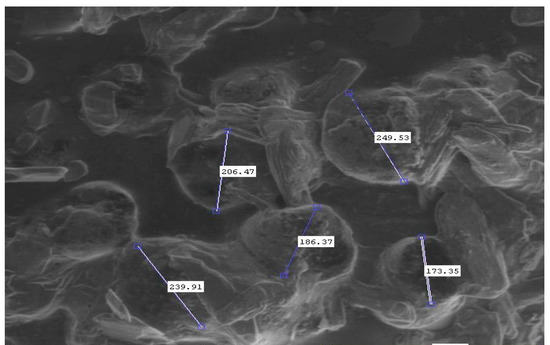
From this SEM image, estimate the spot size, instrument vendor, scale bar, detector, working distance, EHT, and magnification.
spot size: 3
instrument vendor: FEI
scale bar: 400000 nm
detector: LFD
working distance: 8.8 mm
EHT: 20 kV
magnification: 0.3 K X